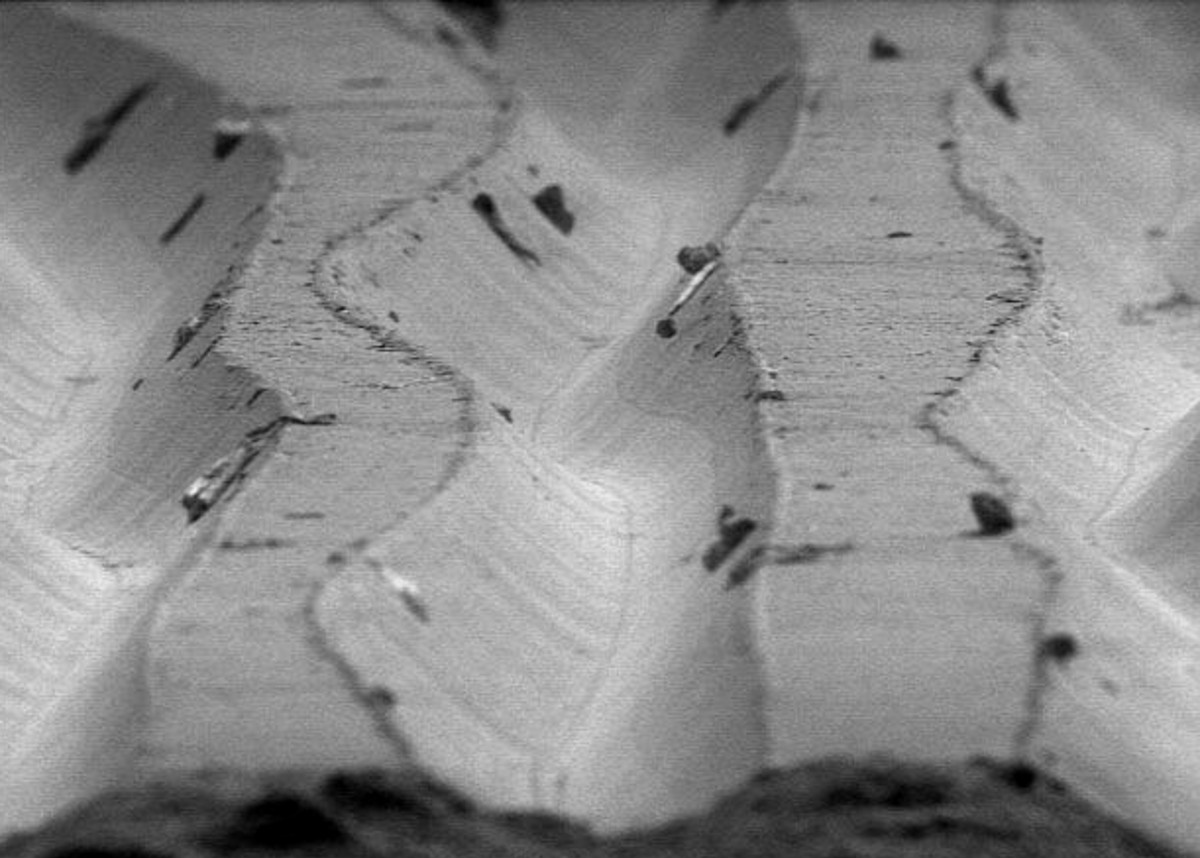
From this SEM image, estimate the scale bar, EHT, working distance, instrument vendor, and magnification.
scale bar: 50000 nm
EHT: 5 kV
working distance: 27 mm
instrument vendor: other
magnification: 0.5 K X